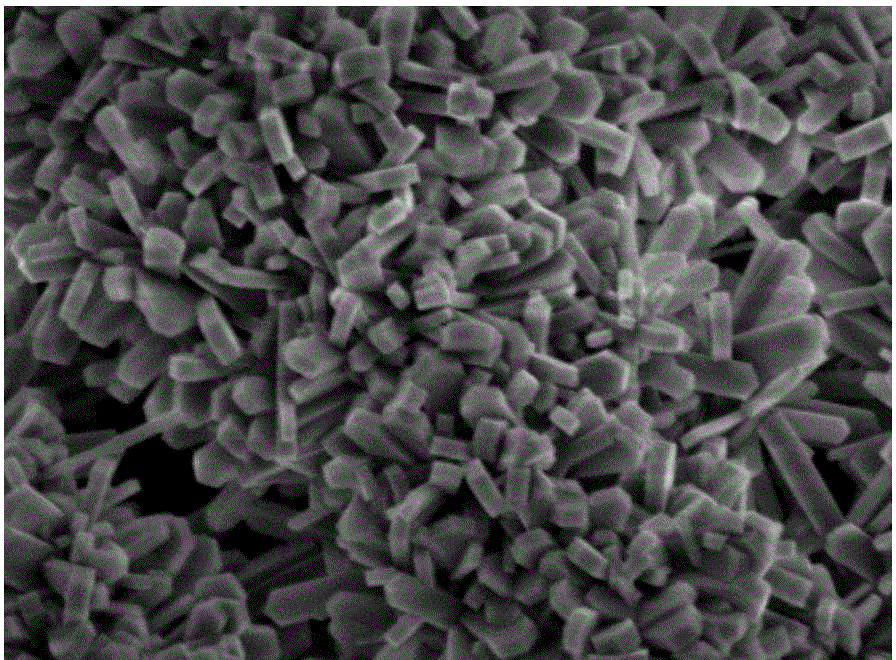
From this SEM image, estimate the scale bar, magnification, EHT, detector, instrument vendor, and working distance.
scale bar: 1000 nm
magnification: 20 K X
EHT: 3 kV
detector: SEI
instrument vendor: JEOL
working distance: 9.3 mm